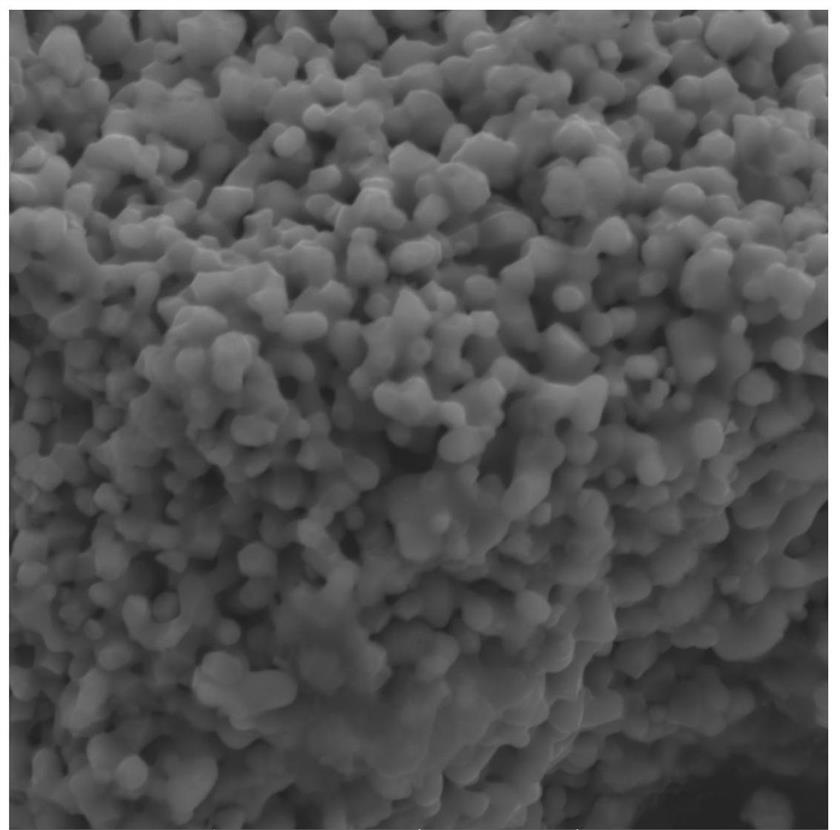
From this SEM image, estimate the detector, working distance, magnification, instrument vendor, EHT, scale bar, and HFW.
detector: SE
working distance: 5.08 mm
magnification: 20 K X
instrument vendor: Tescan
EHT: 10 kV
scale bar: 2000 nm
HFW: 10.4 µm